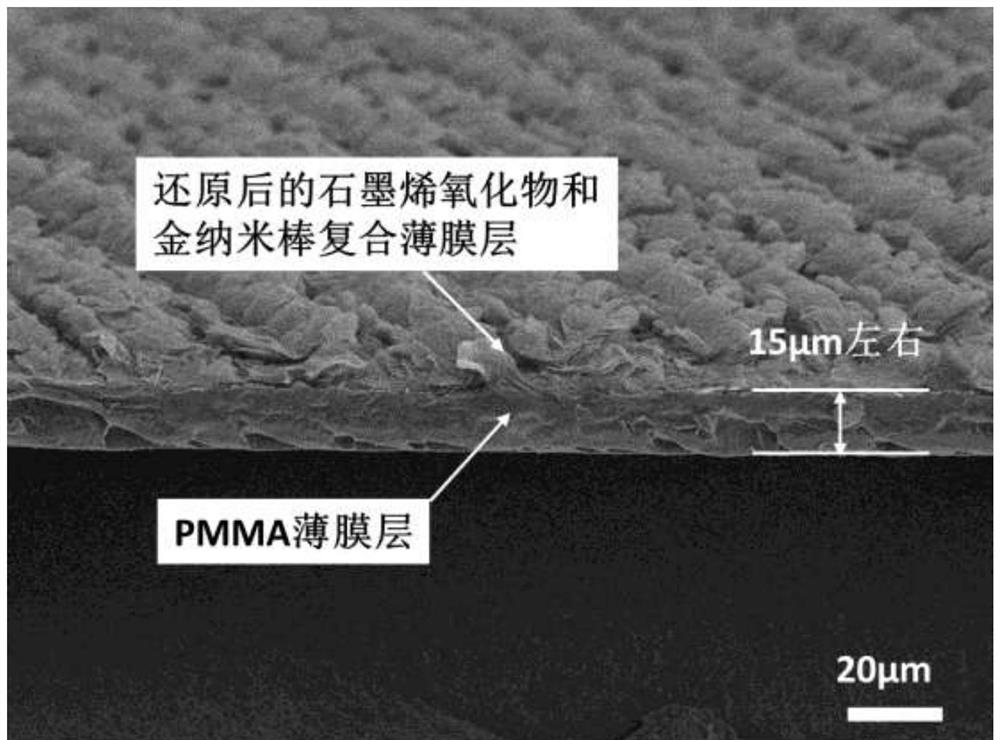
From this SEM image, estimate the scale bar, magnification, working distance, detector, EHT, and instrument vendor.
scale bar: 10000 nm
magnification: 0.6 K X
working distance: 20.2 mm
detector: LEI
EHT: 5 kV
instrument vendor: JEOL